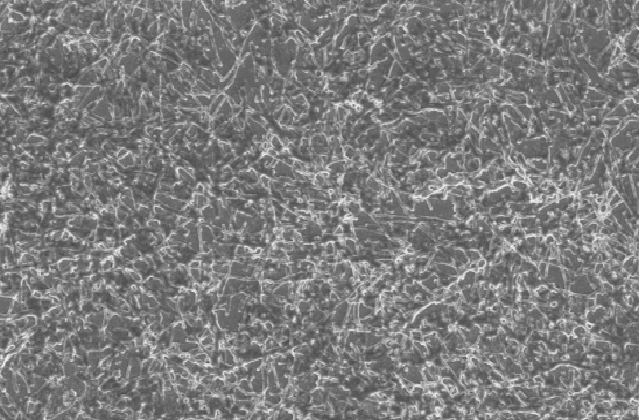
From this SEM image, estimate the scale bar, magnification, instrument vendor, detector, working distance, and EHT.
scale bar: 20000 nm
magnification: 2 K X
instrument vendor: Zeiss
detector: SE1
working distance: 12.5 mm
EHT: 7 kV